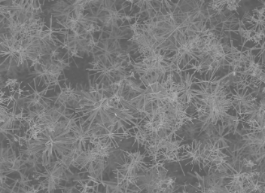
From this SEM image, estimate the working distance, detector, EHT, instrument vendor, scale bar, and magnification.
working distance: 11 mm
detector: SEI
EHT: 10 kV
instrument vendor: JEOL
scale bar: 10000 nm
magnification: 1 K X